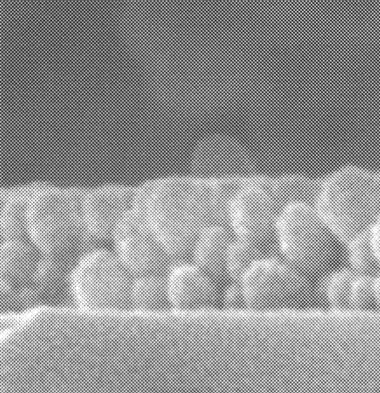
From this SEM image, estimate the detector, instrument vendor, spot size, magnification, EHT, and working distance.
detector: TLD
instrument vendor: FEI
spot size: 2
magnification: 200 K X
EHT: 5 kV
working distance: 4.4 mm